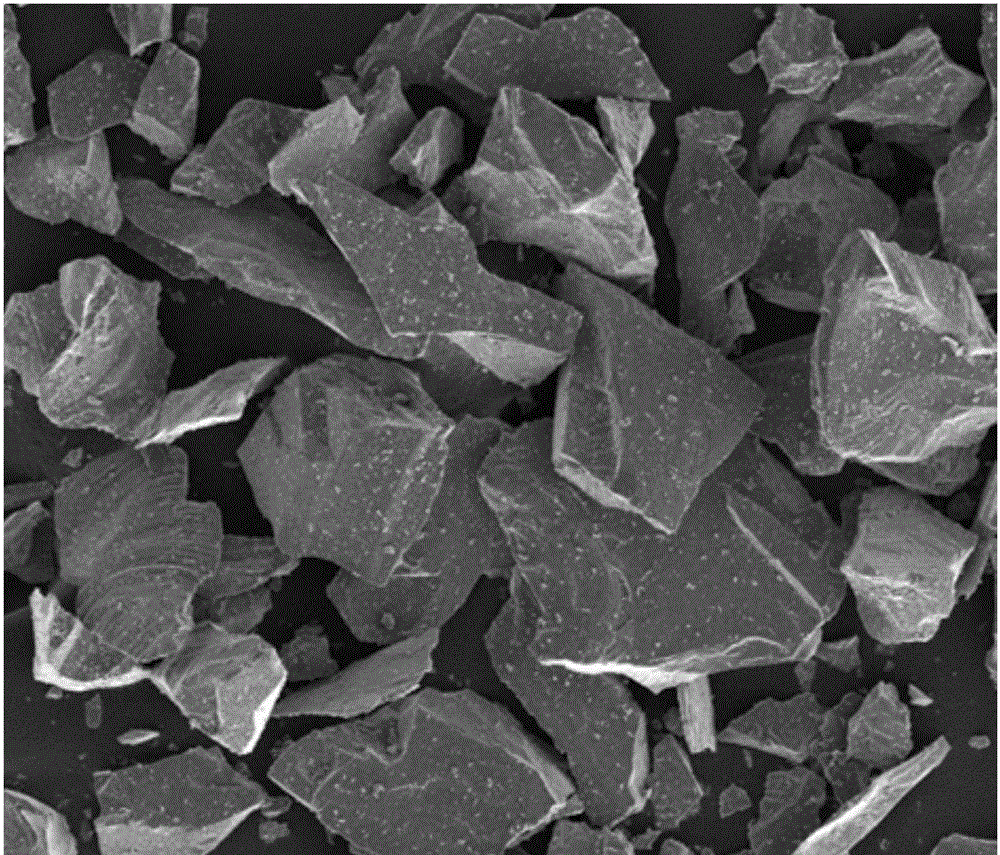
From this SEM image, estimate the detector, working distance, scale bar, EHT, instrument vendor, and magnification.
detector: ETD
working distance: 5.7 mm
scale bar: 200000 nm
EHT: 10 kV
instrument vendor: FEI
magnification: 0.2 K X